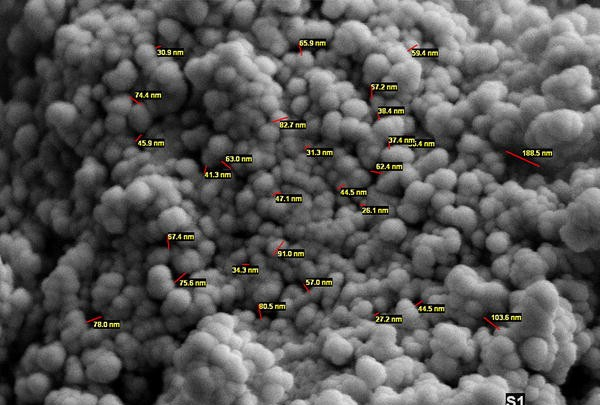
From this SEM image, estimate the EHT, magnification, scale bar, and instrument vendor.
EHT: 25 kV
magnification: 40 K X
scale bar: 1000 nm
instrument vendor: other